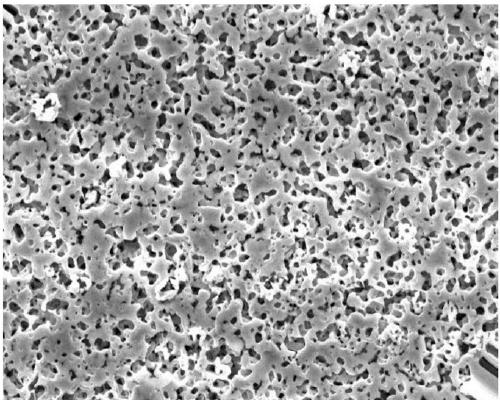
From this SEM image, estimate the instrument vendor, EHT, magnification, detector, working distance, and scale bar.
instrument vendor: JEOL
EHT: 10 kV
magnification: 5 K X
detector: SEI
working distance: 8.8 mm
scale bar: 1000 nm